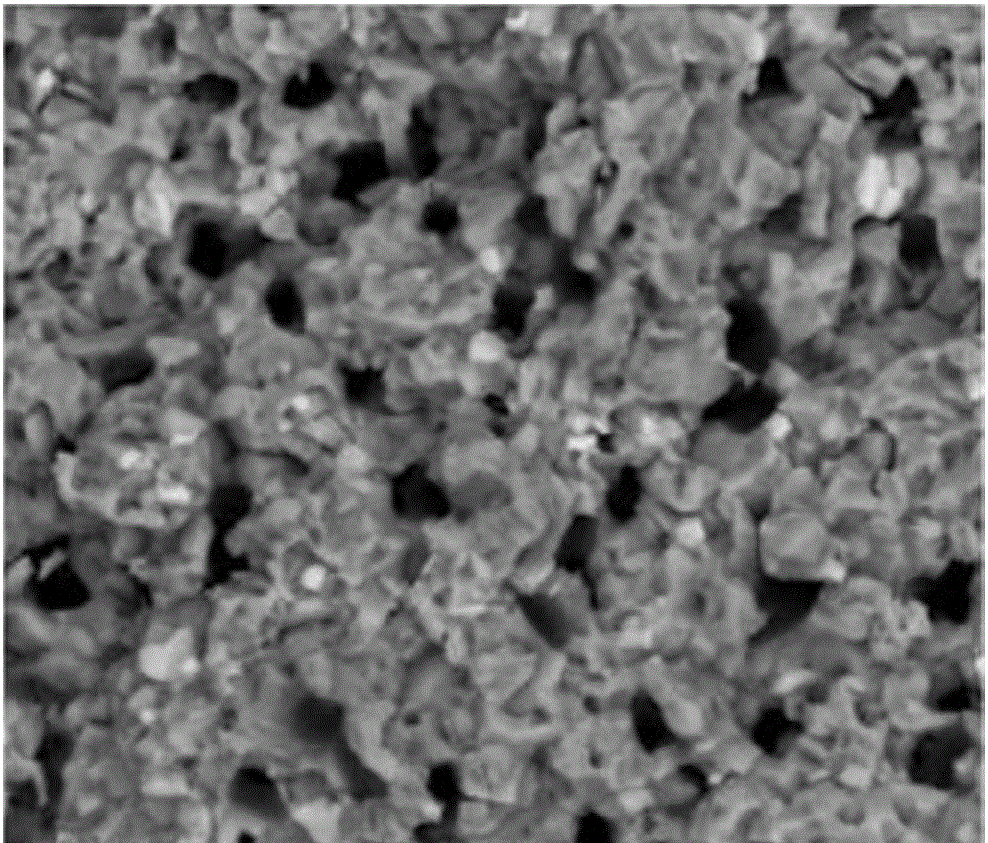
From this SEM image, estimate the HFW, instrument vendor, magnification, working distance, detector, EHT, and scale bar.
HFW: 59.7 µm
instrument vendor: FEI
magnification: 5 K X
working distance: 10.5 mm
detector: BSED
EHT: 20 kV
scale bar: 20000 nm